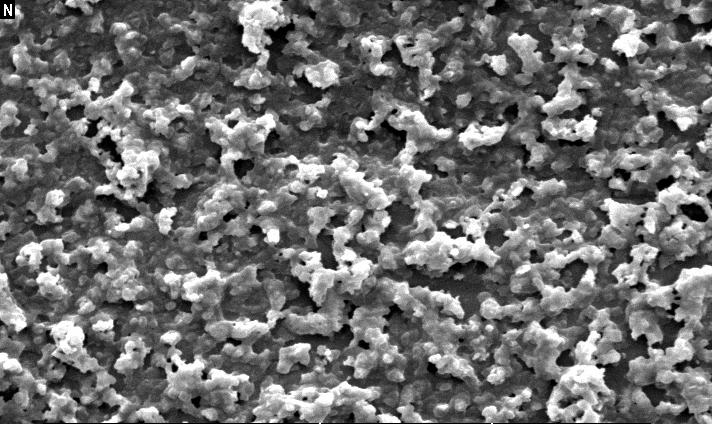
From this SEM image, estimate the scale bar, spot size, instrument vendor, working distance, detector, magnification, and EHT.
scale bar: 10000 nm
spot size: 4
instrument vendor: FEI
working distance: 10 mm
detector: SE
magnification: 3 K X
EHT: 10 kV